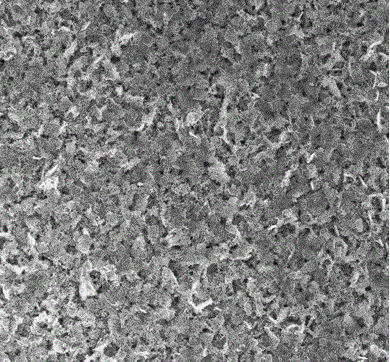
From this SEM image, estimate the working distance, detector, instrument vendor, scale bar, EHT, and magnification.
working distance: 5.2 mm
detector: TLD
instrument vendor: FEI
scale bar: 5000 nm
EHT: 15 kV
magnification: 10 K X